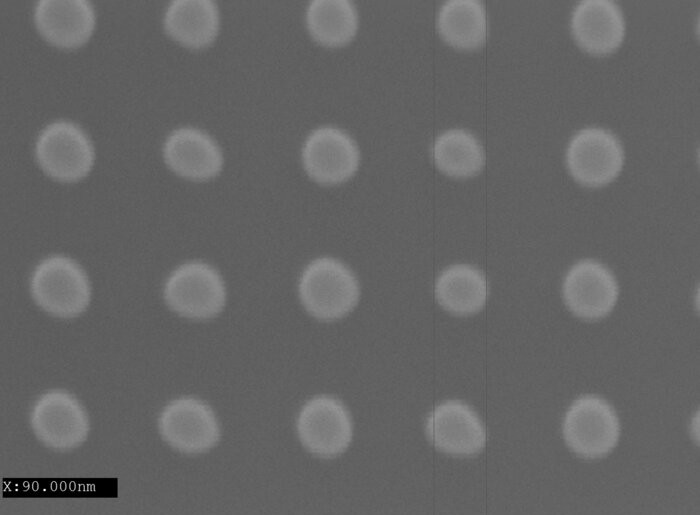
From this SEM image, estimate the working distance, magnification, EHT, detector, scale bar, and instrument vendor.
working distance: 10.2 mm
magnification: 100 K X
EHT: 10 kV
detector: LED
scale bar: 100 nm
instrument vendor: JEOL